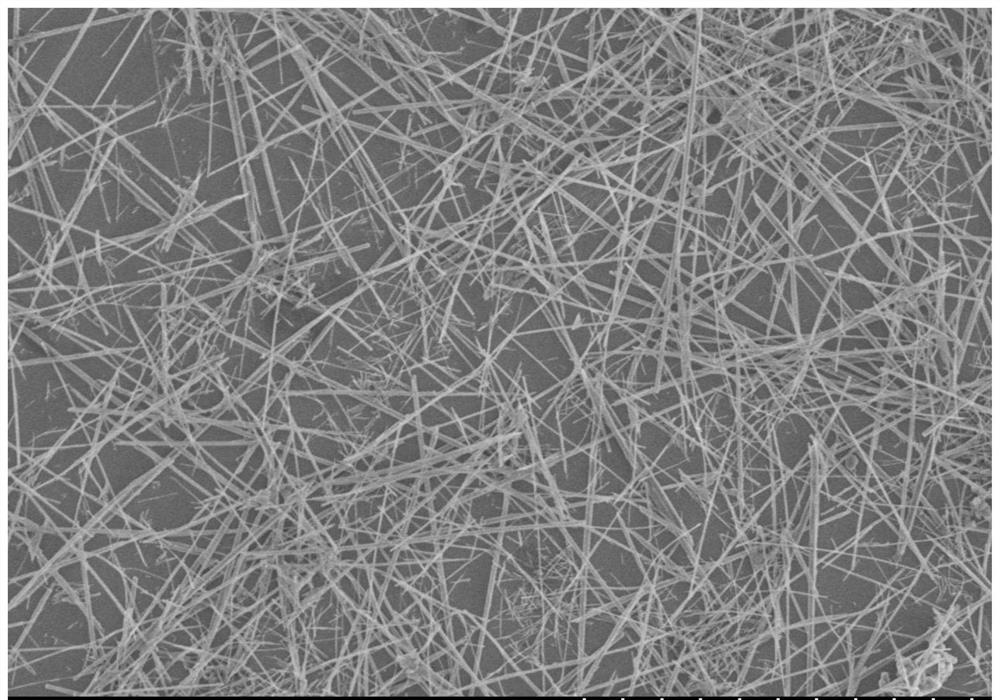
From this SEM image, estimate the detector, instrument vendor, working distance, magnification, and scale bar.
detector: SE(M)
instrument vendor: Hitachi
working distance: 9.1 mm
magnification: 5 K X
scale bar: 10000 nm